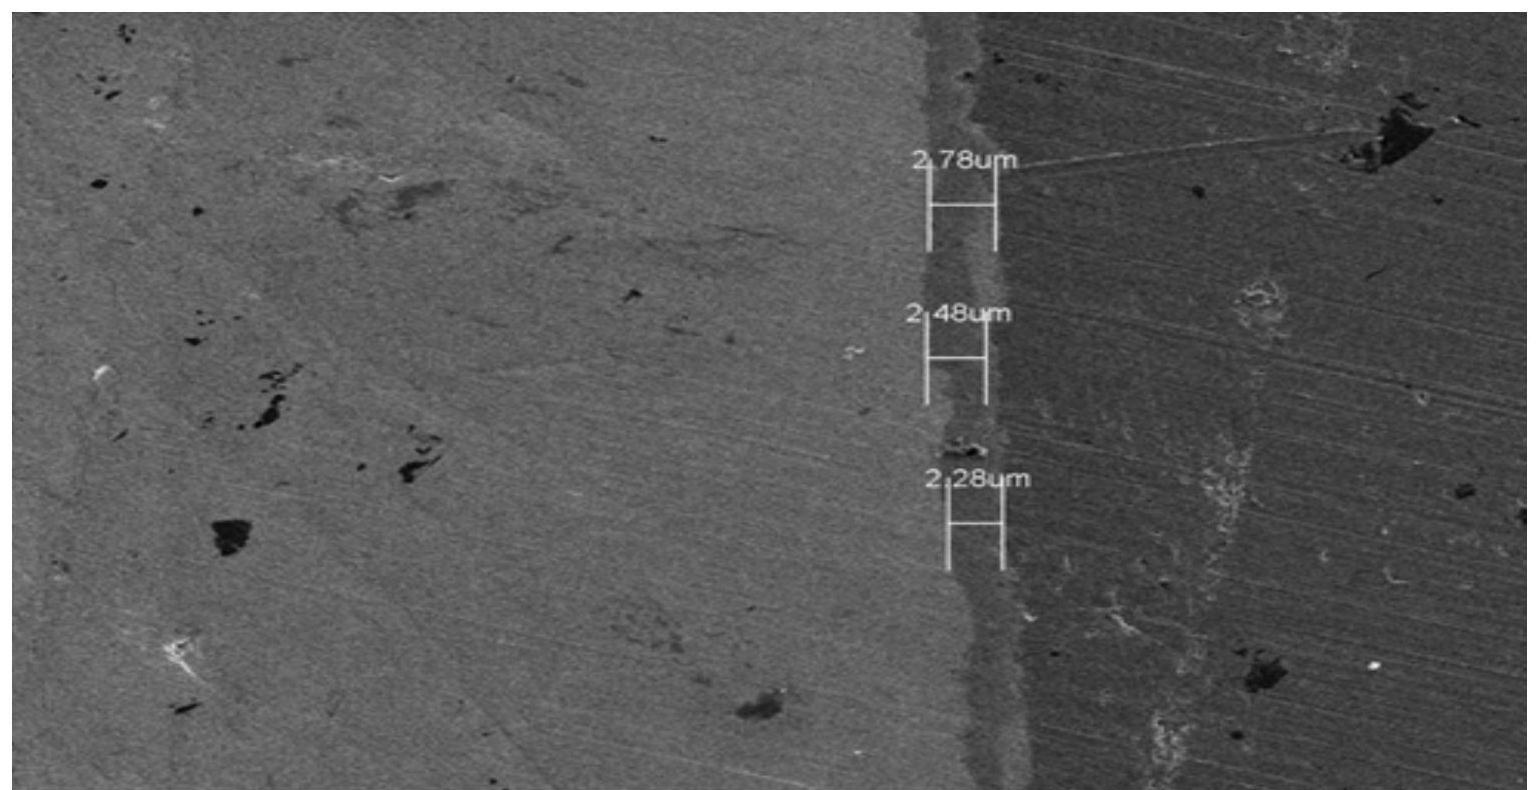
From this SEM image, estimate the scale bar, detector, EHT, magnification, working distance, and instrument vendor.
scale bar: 20000 nm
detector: SE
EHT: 5 kV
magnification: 2 K X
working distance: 7 mm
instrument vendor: Hitachi